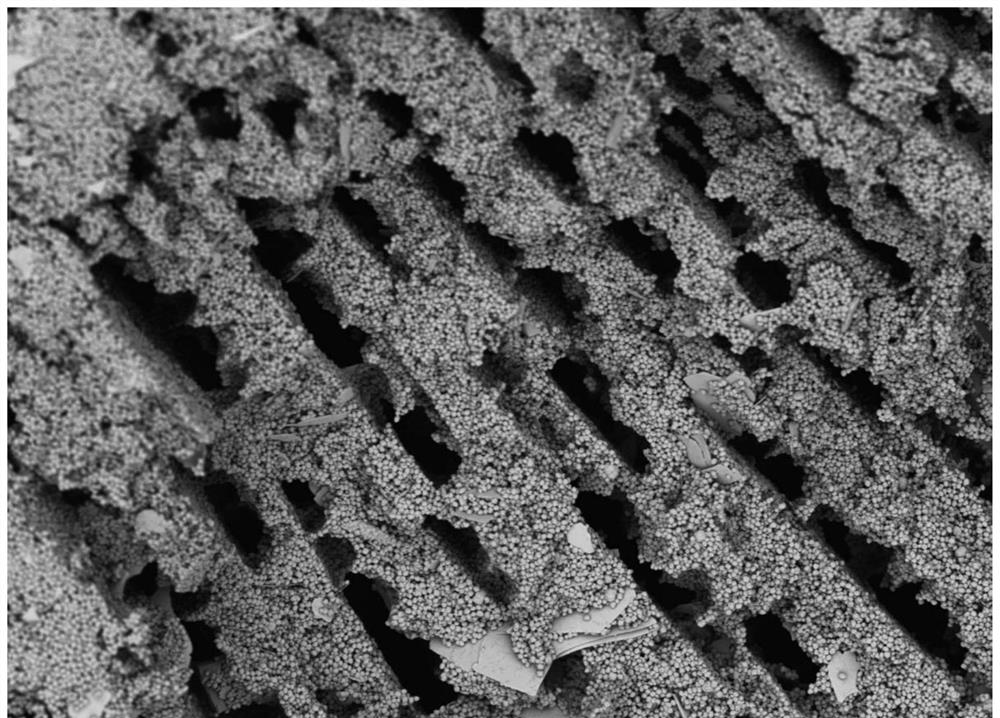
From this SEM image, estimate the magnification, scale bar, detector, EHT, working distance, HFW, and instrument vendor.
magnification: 2 K X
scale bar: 80000 nm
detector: BSD Full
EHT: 5 kV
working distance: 6.829 mm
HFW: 259 µm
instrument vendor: Thermo